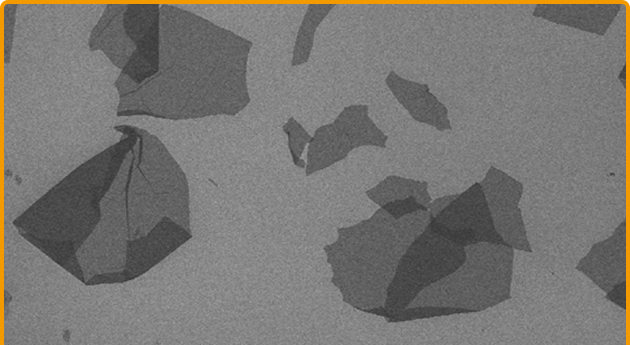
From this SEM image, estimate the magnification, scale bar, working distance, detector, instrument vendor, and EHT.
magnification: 0.3 K X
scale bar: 100000 nm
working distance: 10.8 mm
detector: SE(M)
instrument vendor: Hitachi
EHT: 3 kV